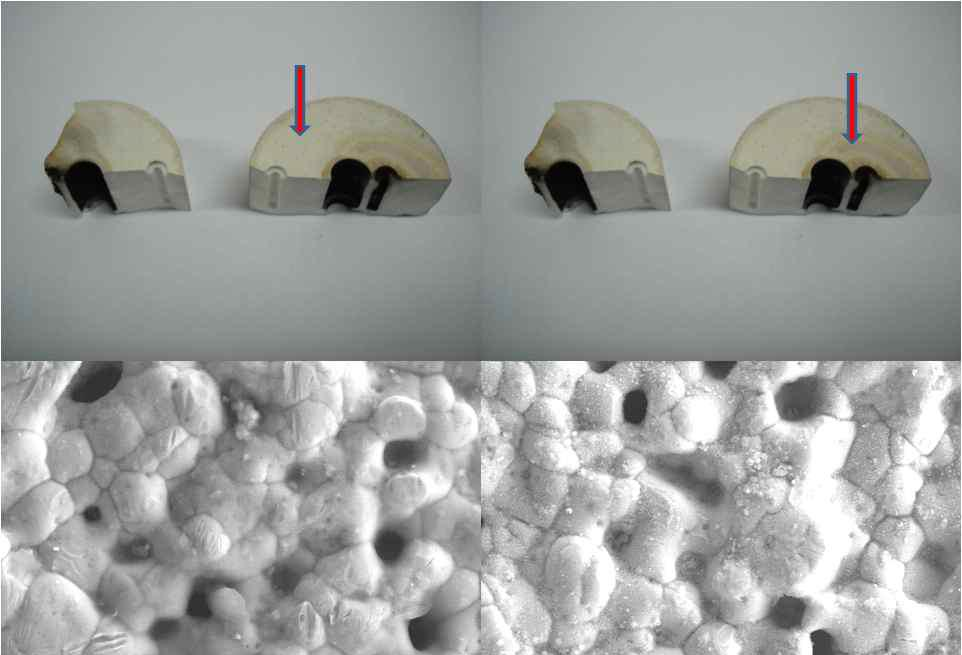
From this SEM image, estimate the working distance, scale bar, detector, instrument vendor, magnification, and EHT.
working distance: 12 mm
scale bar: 10000 nm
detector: SE(M)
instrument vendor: Hitachi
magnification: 3 K X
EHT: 15 kV